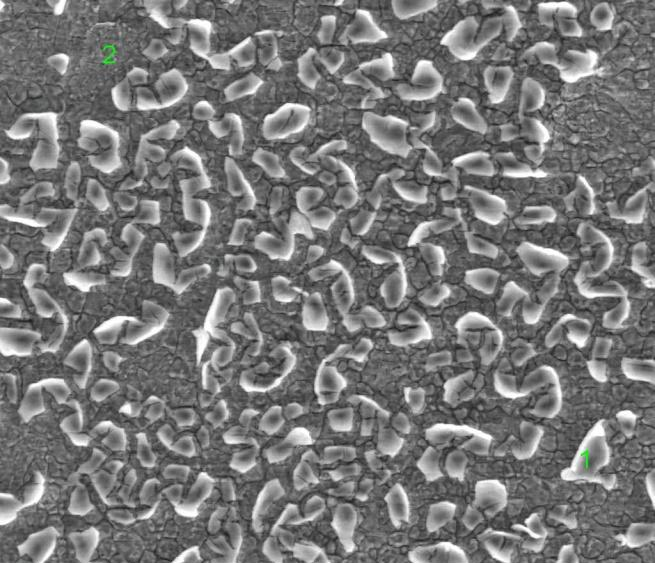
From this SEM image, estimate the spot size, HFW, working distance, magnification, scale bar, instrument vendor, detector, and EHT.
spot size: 4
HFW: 149 µm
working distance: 5.3 mm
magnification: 2 K X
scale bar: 50000 nm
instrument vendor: FEI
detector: LVD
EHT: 18 kV